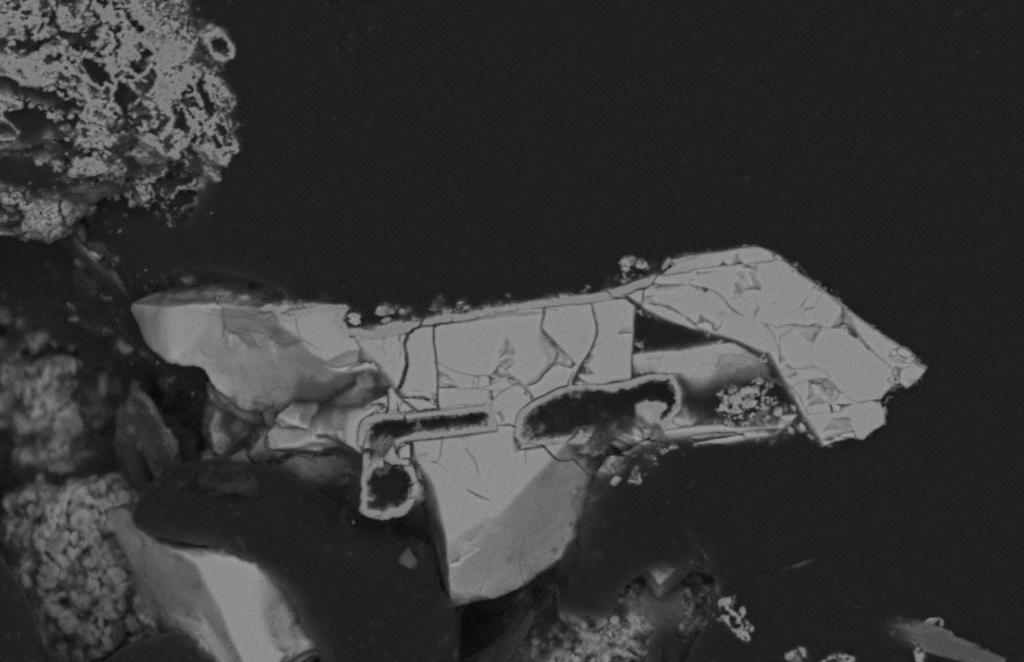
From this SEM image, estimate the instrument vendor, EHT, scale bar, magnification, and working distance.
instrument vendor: Hitachi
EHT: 15 kV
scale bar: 50000 nm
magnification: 0.85 K X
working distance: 11 mm